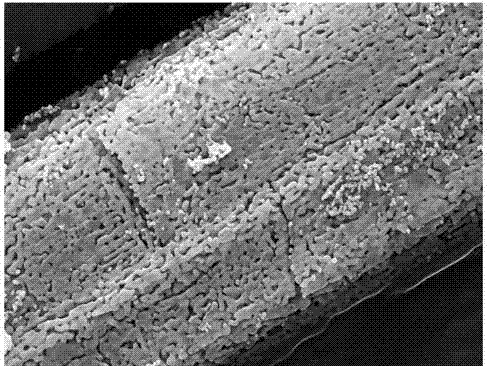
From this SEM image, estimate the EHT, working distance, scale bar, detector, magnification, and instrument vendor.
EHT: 15 kV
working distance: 10.1 mm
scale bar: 1000 nm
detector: SE2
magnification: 10 K X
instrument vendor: Zeiss